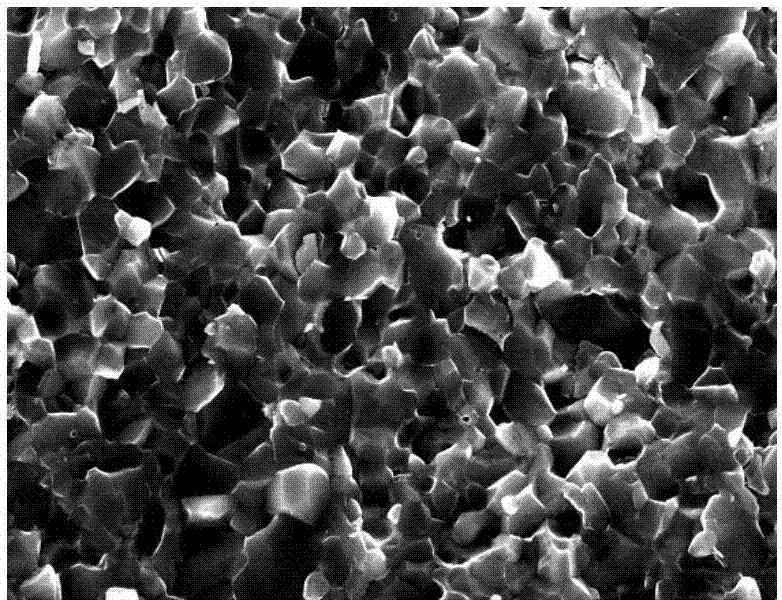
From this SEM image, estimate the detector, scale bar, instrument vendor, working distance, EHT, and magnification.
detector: SE(M)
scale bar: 5000 nm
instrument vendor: Hitachi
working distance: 9.6 mm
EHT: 15 kV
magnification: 10 K X